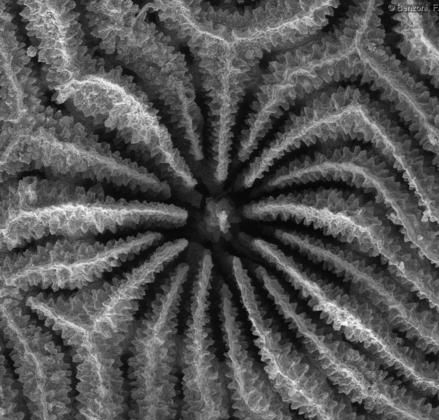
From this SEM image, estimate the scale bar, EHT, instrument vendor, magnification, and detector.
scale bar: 1000000 nm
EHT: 20 kV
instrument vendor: Tescan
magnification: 0.228 K X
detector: SE Detector